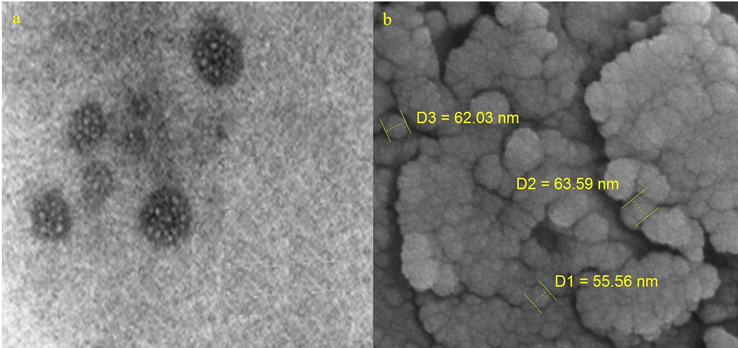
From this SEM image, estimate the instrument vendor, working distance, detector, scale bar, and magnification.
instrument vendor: Tescan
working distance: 5.01 mm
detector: InBeam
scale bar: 200 nm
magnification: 200 K X